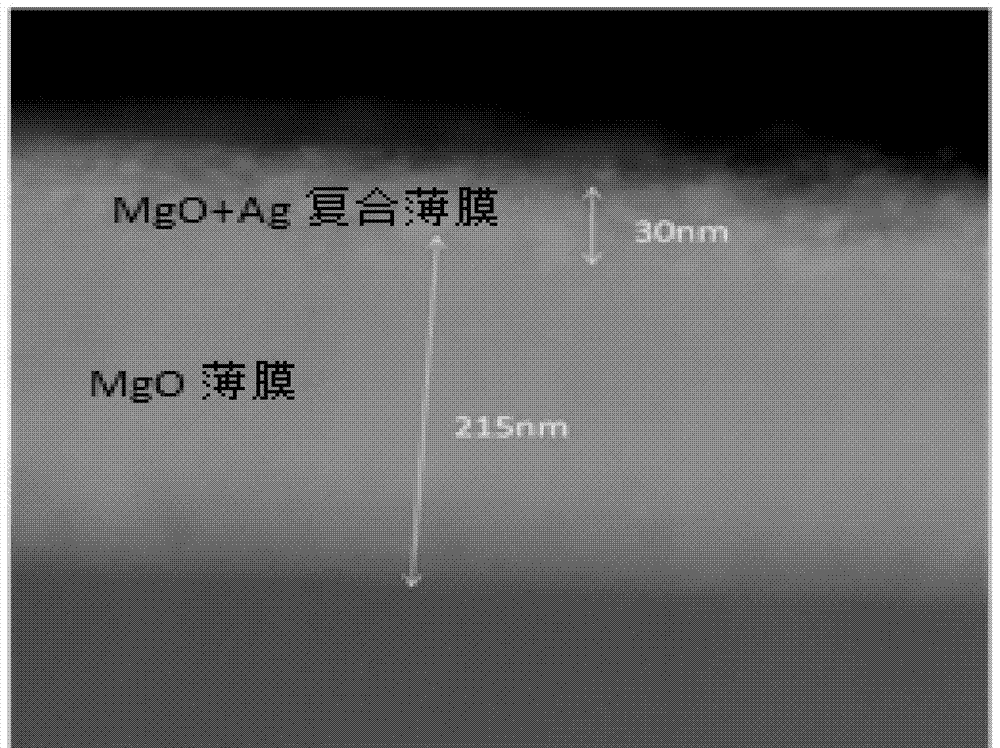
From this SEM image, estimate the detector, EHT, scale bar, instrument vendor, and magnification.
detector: STEM DF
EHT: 200 kV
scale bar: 100 nm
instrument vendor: JEOL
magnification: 300 K X